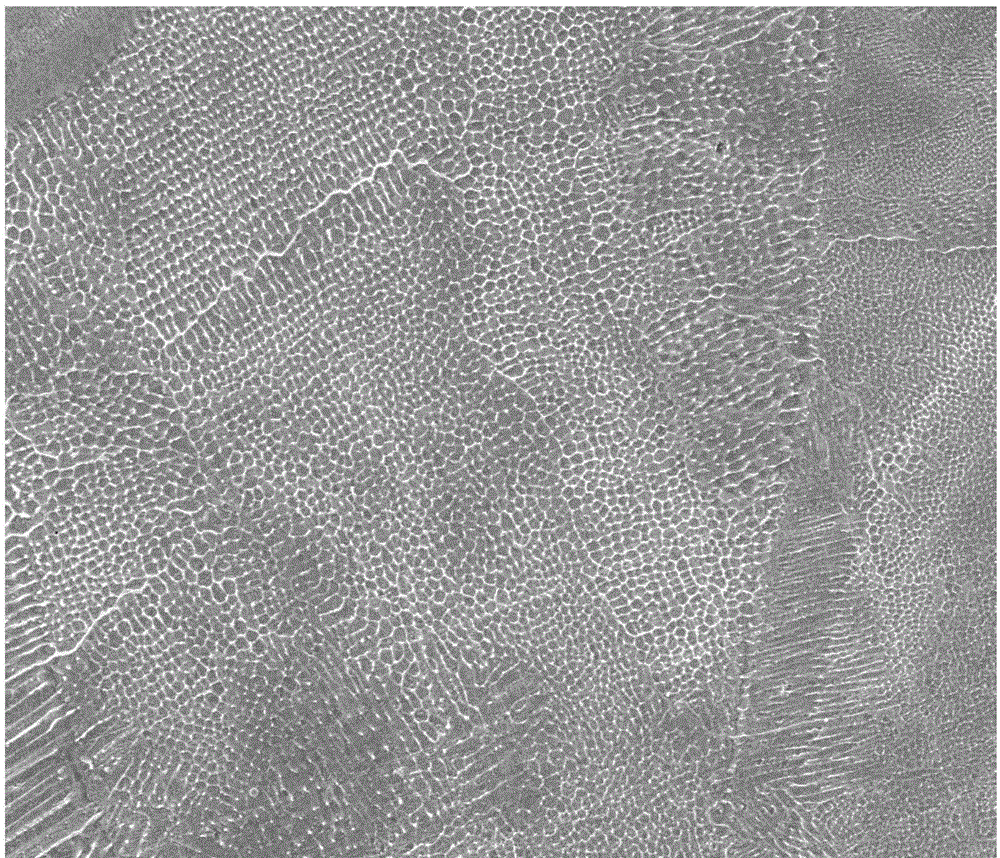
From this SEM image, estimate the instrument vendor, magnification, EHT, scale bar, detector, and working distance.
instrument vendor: FEI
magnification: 5 K X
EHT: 30 kV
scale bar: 20000 nm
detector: ETD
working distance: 9.6 mm